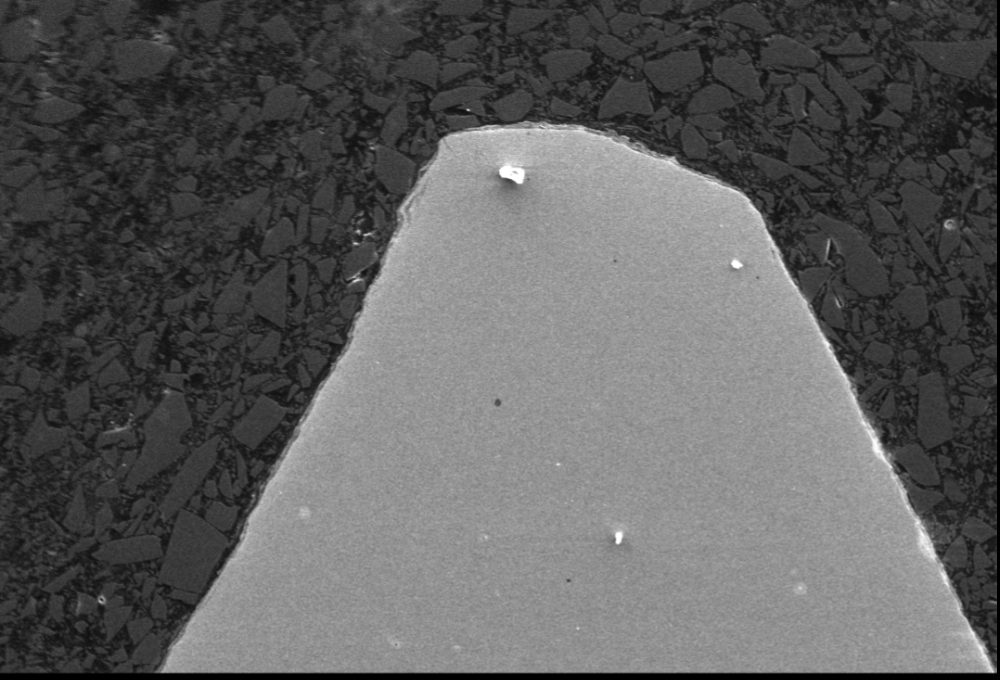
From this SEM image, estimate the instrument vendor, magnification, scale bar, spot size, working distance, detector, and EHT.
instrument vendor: other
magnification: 0.2 K X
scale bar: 100000 nm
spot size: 8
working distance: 8.4 mm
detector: SE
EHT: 15 kV